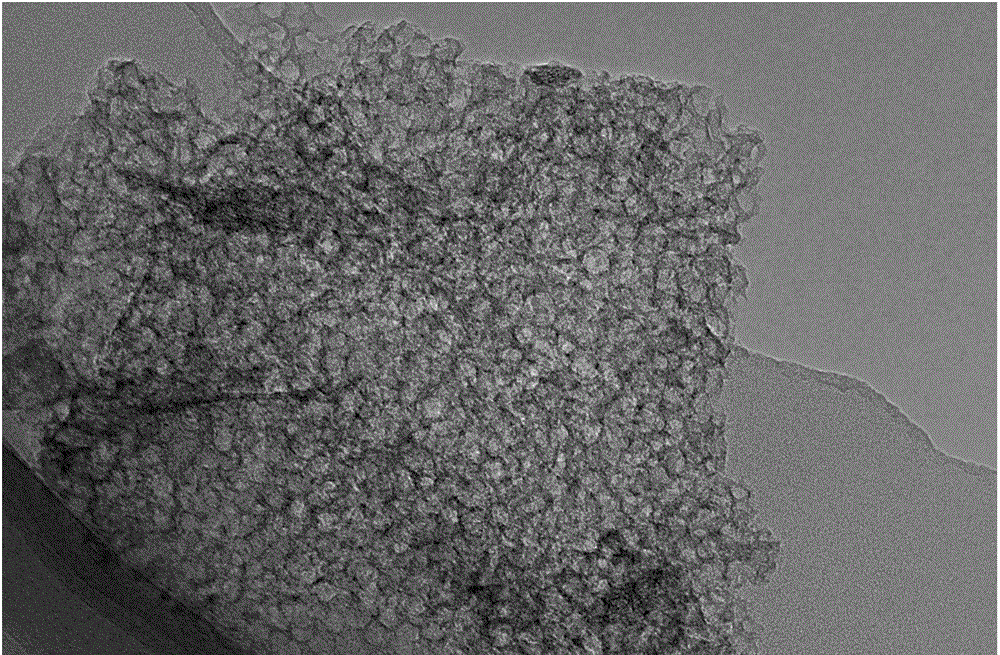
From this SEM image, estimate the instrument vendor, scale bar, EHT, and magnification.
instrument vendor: JEOL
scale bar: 100 nm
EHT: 200 kV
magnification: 80 K X